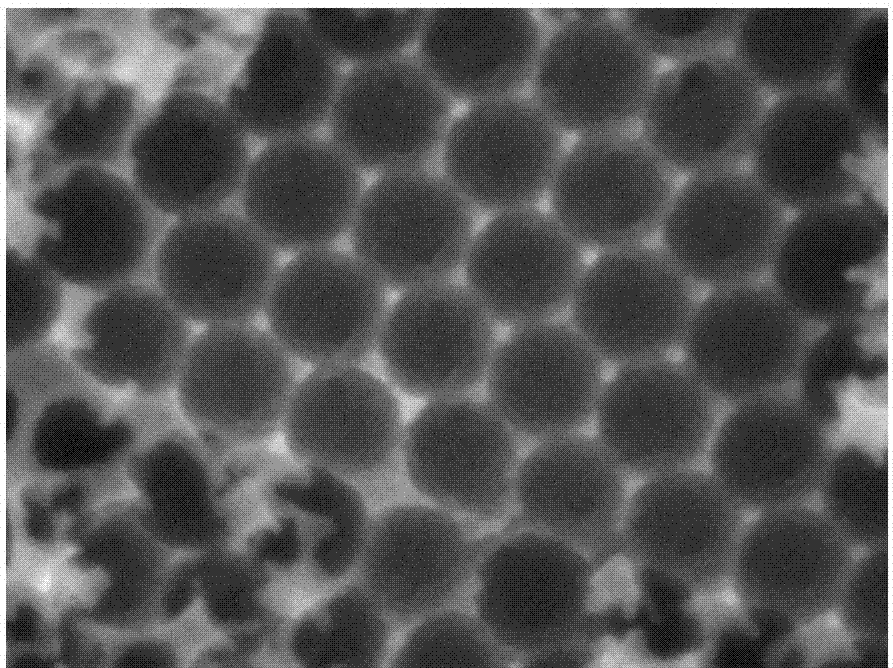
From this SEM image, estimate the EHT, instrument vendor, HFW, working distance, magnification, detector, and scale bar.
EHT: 5 kV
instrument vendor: Tescan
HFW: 0.843 µm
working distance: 6 mm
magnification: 300 K X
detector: InBeam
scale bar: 200 nm